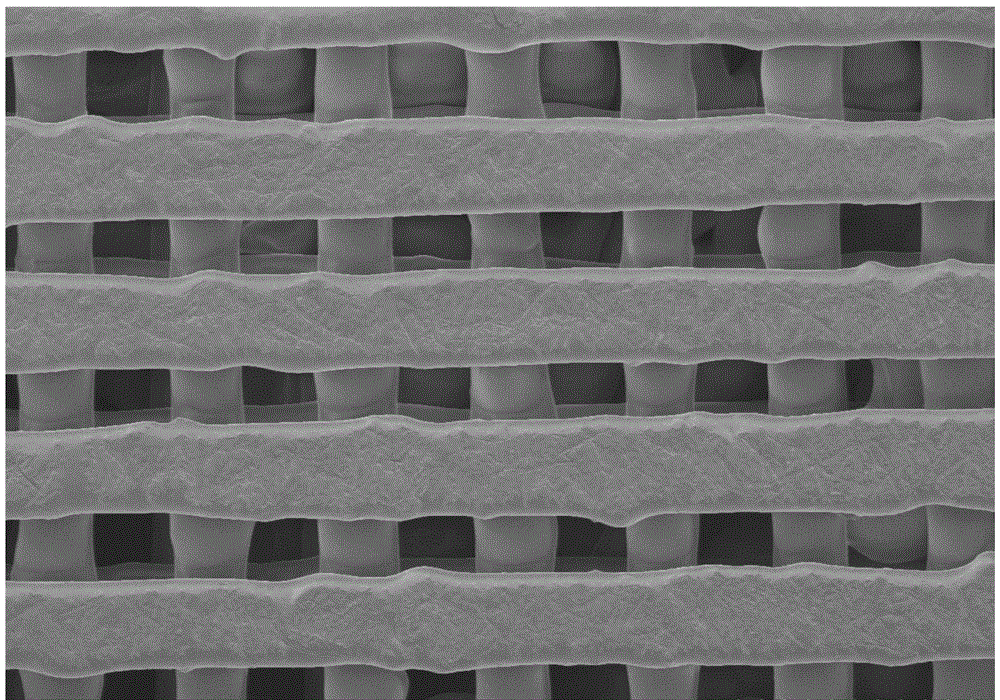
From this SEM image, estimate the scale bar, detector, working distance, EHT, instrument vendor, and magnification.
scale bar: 1000000 nm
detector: LM(UL)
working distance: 8 mm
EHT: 1.5 kV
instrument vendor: Hitachi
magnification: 0.03 K X